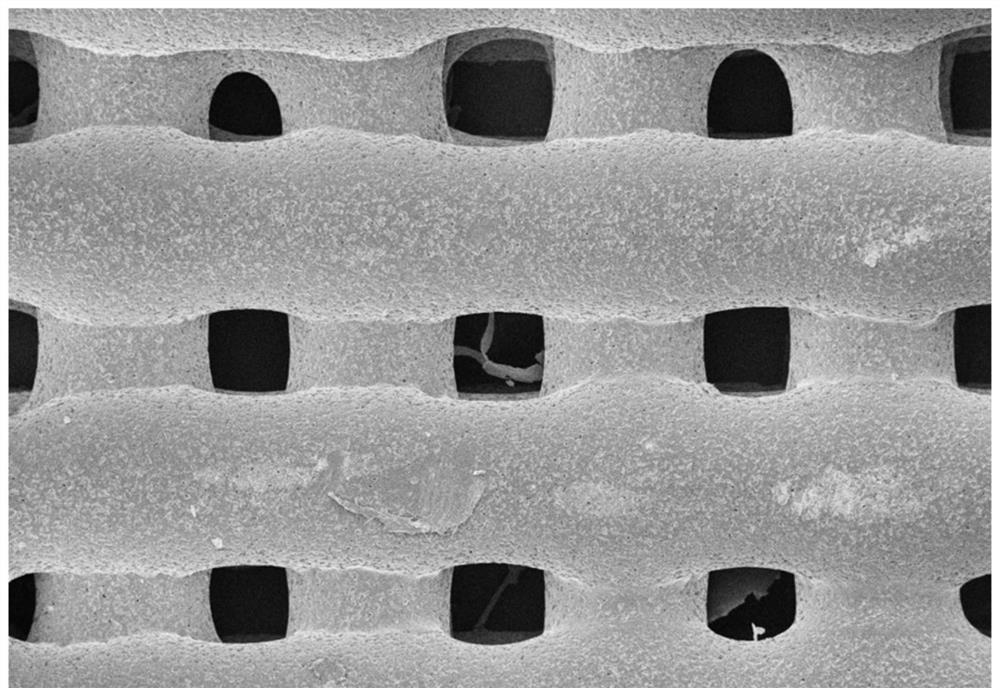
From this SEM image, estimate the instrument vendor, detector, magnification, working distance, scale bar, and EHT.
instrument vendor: Zeiss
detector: InLens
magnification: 0.05 K X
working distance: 6.6 mm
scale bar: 100000 nm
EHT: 5 kV